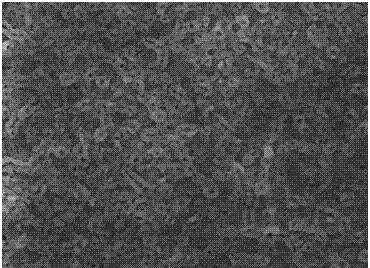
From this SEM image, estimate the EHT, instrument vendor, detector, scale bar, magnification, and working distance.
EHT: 20 kV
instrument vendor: Tescan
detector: SE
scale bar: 20000 nm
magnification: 3 K X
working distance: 15.48 mm